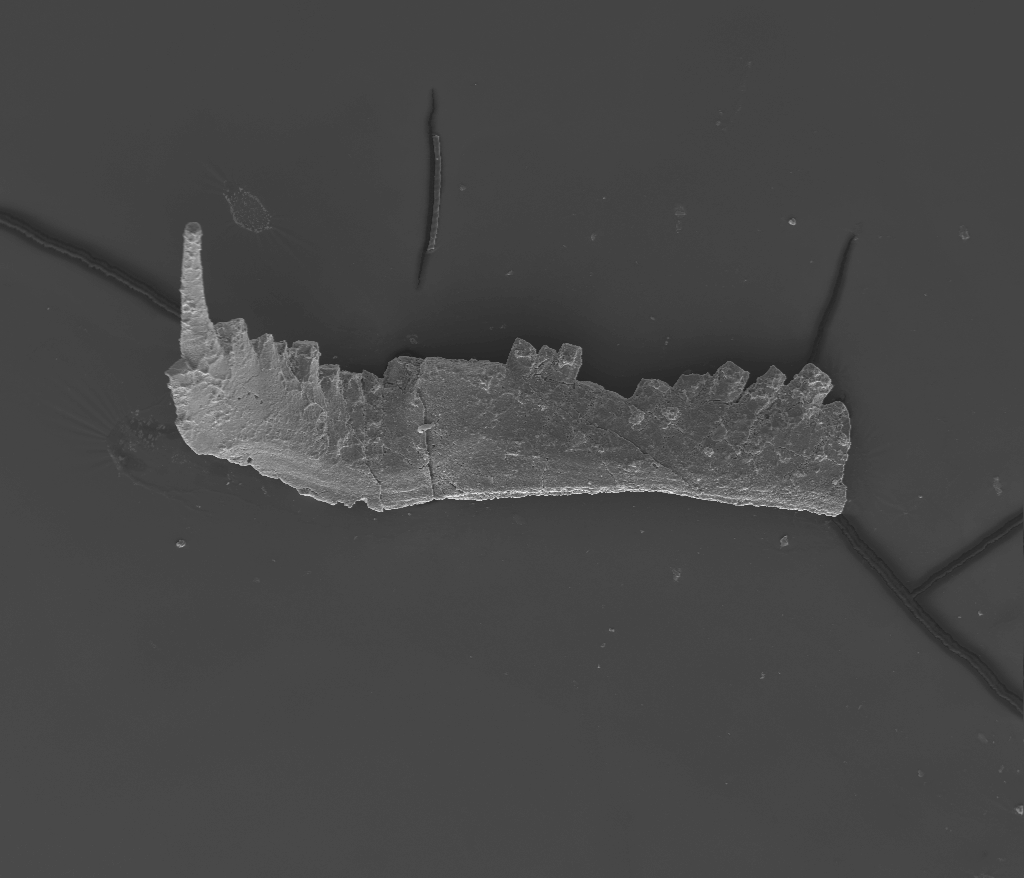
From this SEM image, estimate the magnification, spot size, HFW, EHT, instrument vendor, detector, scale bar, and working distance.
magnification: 0.16 K X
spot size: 5.5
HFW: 1690 µm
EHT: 20 kV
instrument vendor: FEI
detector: ETD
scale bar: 400000 nm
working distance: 10.2 mm